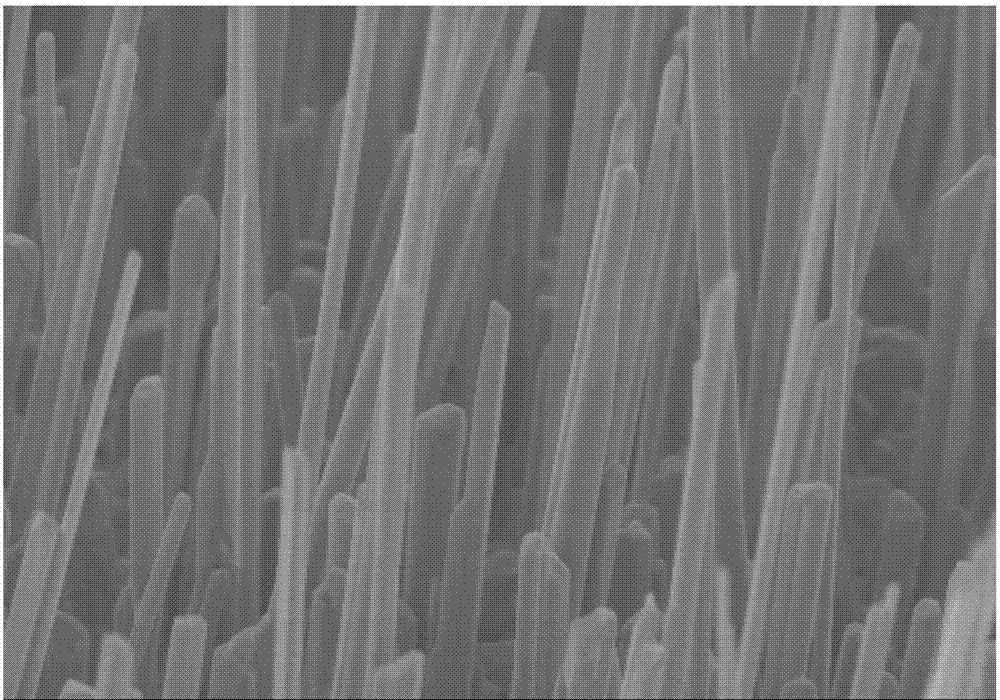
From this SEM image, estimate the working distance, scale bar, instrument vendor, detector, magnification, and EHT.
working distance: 10.1 mm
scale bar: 5000 nm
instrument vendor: Hitachi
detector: SE(M)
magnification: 10 K X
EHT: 5 kV